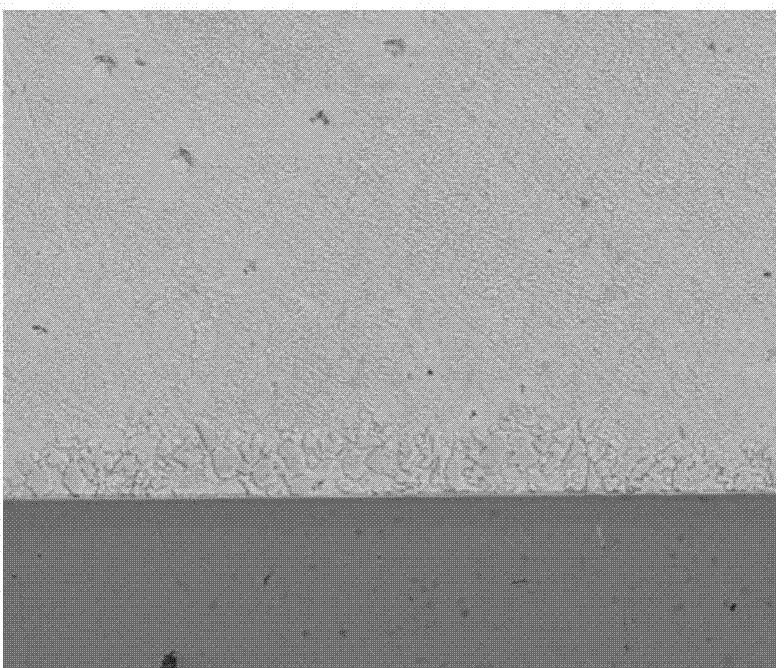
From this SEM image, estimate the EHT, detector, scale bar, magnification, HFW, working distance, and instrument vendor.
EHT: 18 kV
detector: BSED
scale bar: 50000 nm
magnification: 1 K X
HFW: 240 µm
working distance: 5.1 mm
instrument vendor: FEI